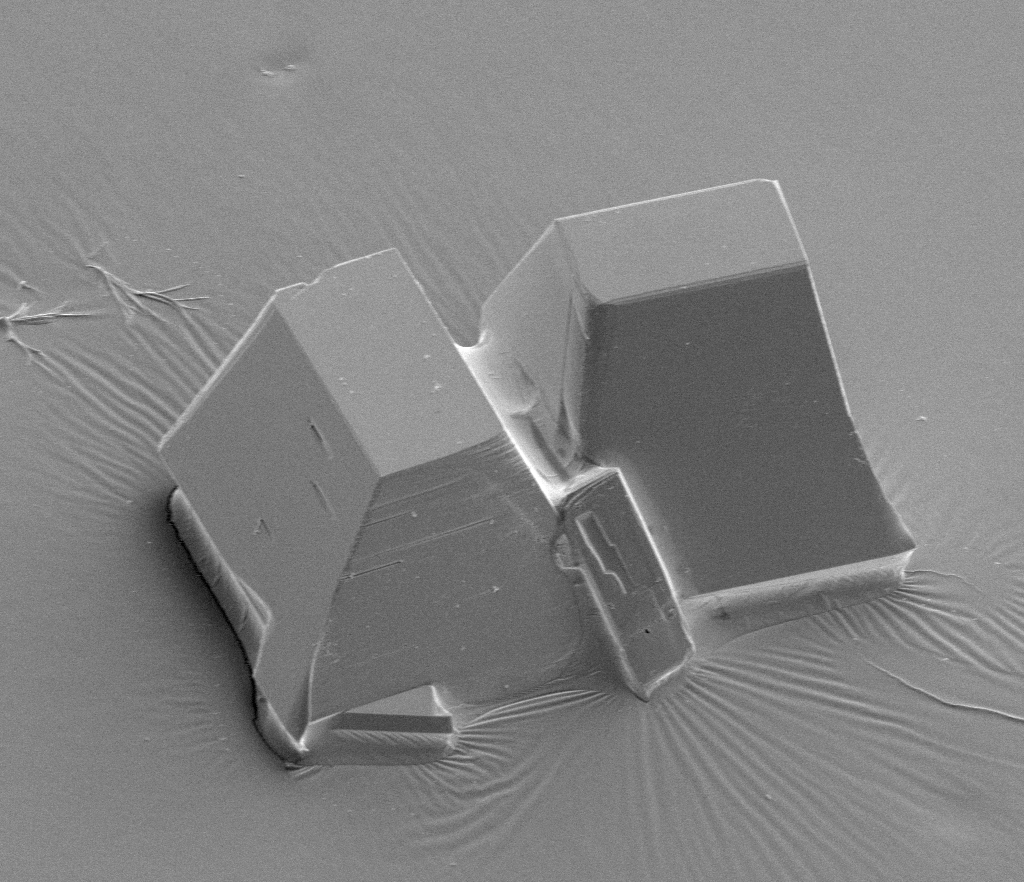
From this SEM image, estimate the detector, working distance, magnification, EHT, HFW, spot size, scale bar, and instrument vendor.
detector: ETD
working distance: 7.7 mm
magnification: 1.748 K X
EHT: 5 kV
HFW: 177 µm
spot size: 5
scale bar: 40000 nm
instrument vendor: FEI